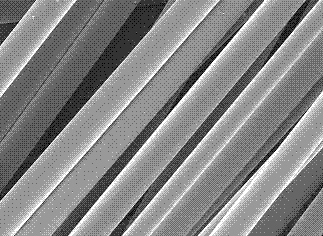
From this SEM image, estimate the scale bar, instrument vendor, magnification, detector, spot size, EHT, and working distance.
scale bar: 20000 nm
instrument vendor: FEI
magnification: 1 K X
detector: SE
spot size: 3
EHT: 10 kV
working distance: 12.1 mm